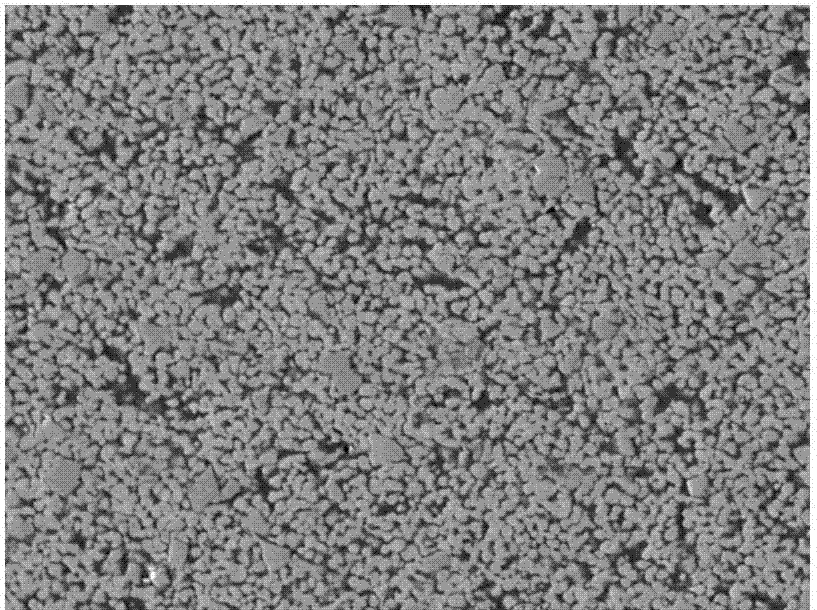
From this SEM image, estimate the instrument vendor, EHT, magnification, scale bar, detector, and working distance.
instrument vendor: JEOL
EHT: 20 kV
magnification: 1 K X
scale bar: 10000 nm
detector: LEI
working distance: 14.5 mm